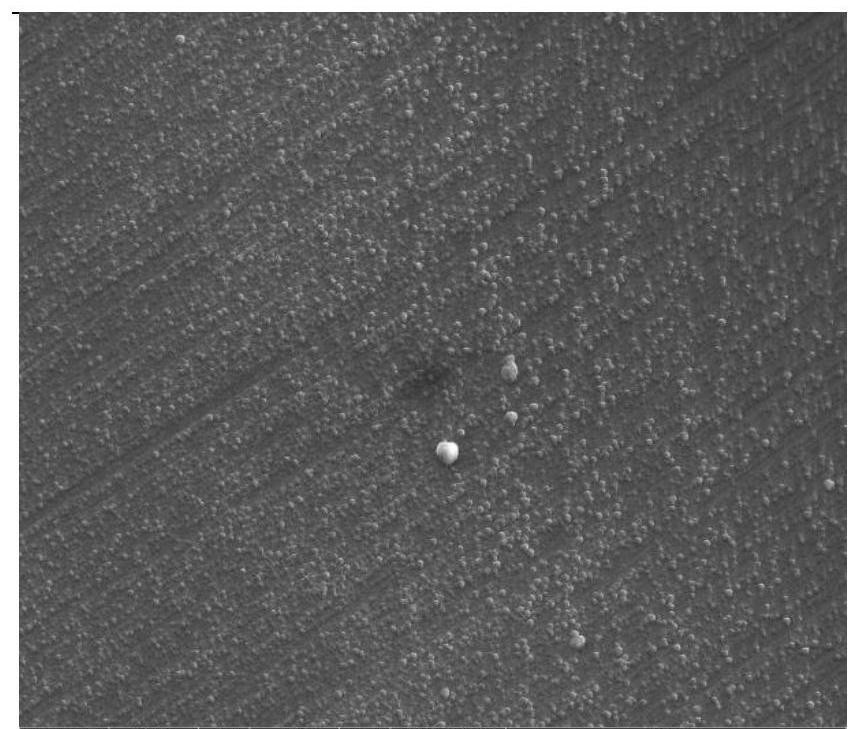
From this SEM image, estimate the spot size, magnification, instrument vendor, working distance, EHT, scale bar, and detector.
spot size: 3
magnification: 10 K X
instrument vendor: FEI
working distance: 10 mm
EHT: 30 kV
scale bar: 10000 nm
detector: ETD